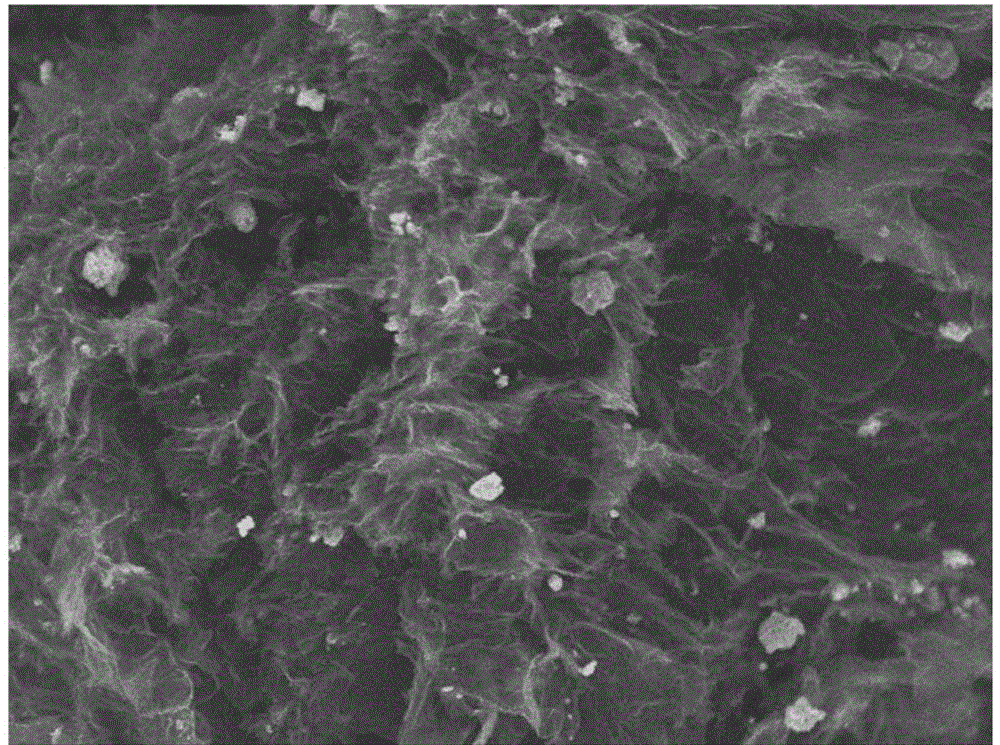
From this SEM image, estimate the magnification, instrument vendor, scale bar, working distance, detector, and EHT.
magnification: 2 K X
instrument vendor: JEOL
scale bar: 10000 nm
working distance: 8.2 mm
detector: SEI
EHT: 10 kV